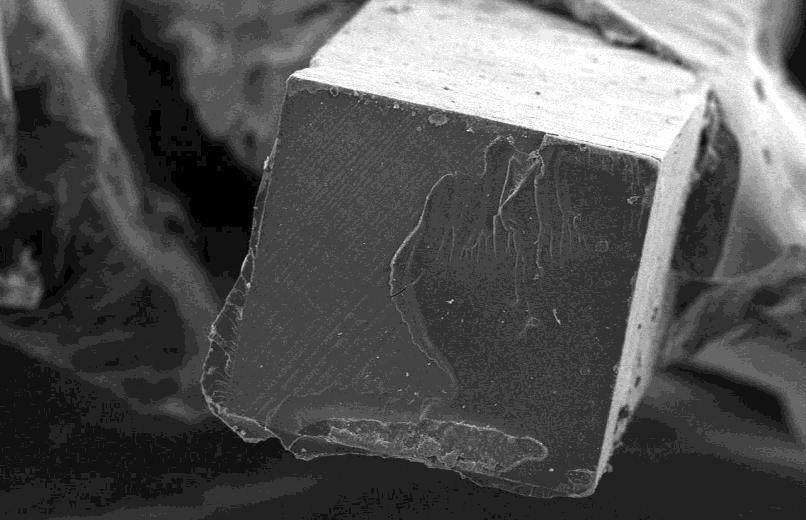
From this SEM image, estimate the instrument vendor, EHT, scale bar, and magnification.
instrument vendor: JEOL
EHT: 15 kV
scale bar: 200000 nm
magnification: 0.06 K X